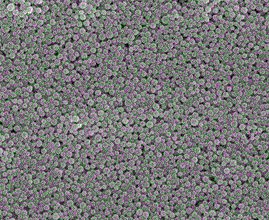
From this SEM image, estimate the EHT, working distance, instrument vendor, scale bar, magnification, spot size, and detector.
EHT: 15 kV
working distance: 9.8 mm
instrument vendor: FEI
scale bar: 5000 nm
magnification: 5 K X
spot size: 3.5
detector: ETD